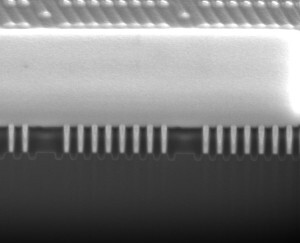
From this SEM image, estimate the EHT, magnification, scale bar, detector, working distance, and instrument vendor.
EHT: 10 kV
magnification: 50 K X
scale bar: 2000 nm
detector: ETD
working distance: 9.8 mm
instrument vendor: FEI